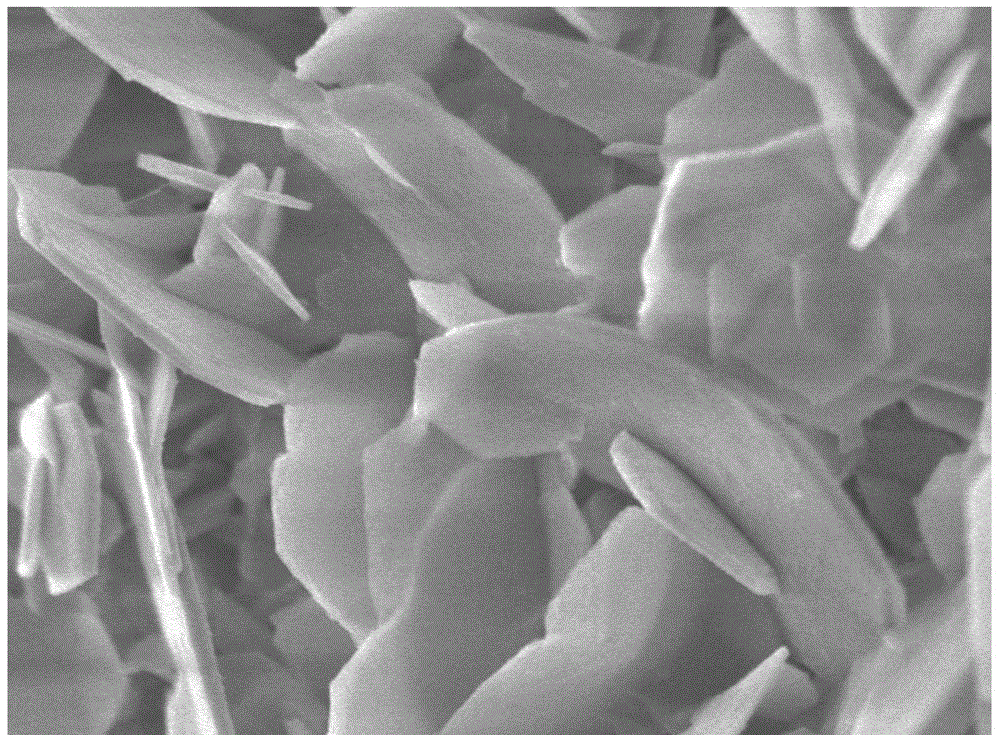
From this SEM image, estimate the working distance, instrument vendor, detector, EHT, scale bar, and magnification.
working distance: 3 mm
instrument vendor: JEOL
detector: UED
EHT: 3 kV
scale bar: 100 nm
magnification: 30 K X